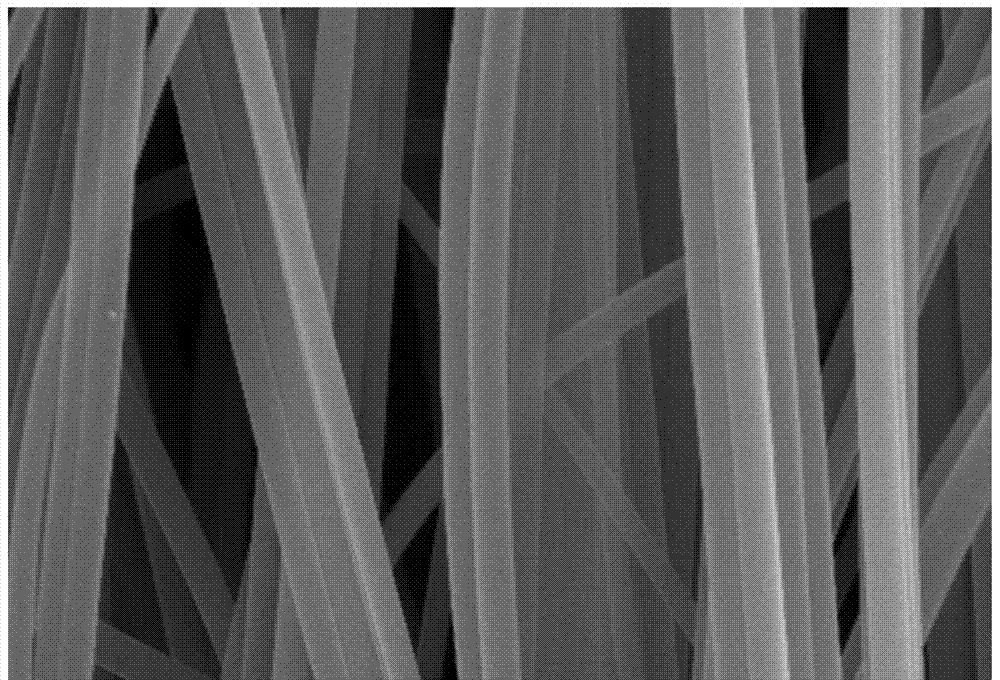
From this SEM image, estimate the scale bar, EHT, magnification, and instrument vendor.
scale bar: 5000 nm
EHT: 20 kV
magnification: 5 K X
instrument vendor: JEOL